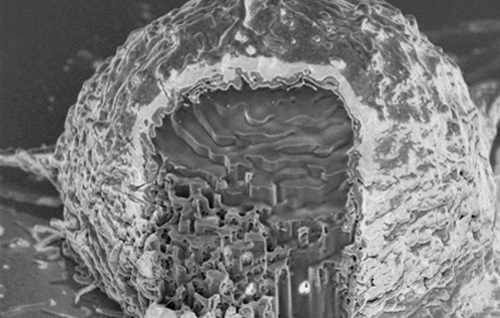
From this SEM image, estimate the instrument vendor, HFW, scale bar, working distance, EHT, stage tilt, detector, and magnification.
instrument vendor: FEI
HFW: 13.8 µm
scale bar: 3000 nm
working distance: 3.9 mm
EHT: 2 kV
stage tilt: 52°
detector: TLD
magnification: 15 K X